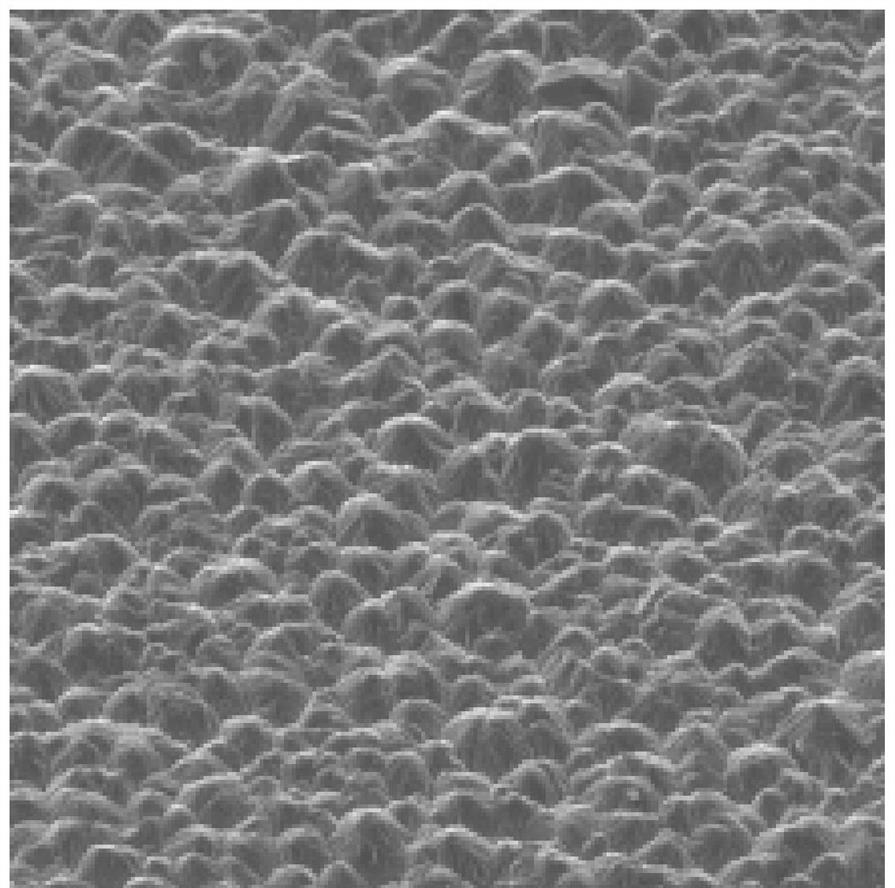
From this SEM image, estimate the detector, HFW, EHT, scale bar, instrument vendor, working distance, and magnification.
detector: SE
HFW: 208 µm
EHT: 20 kV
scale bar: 50000 nm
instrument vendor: Tescan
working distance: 23.75 mm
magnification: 1 K X